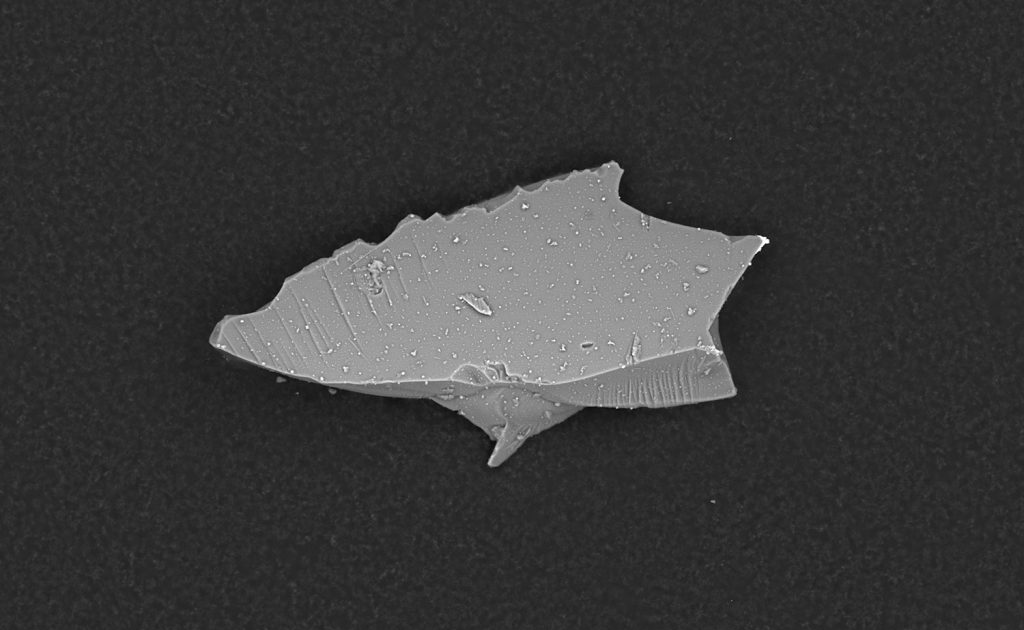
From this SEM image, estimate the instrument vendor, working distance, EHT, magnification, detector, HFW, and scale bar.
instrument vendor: other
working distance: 4.999 mm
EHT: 10 kV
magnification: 3 K X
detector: BSD Full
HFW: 174 µm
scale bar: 50000 nm